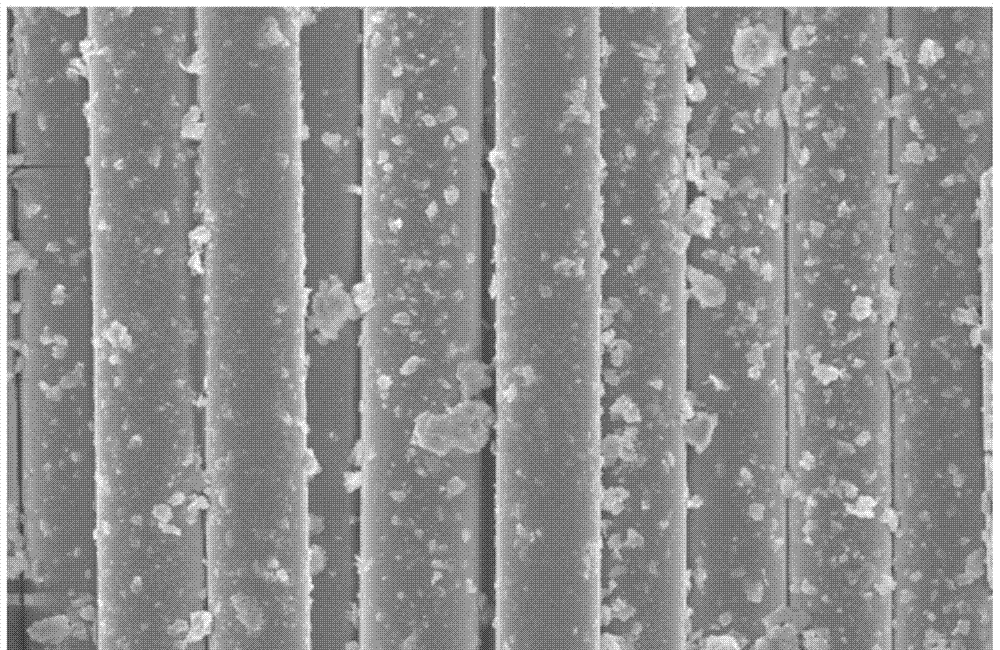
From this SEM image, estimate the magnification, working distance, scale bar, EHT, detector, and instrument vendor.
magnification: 1.3 K X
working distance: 9.1 mm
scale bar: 40000 nm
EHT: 10 kV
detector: SE(U)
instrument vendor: Hitachi